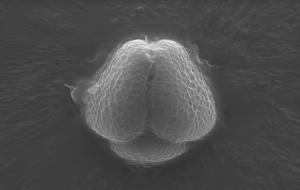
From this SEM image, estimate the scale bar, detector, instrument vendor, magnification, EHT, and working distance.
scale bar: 5000 nm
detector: ETD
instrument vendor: FEI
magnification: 15 K X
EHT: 12.5 kV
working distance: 10.2 mm